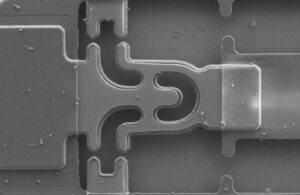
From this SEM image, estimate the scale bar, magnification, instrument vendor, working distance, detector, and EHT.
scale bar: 2000 nm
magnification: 3.5 K X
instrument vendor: Zeiss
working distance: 5 mm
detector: SE2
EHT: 2 kV